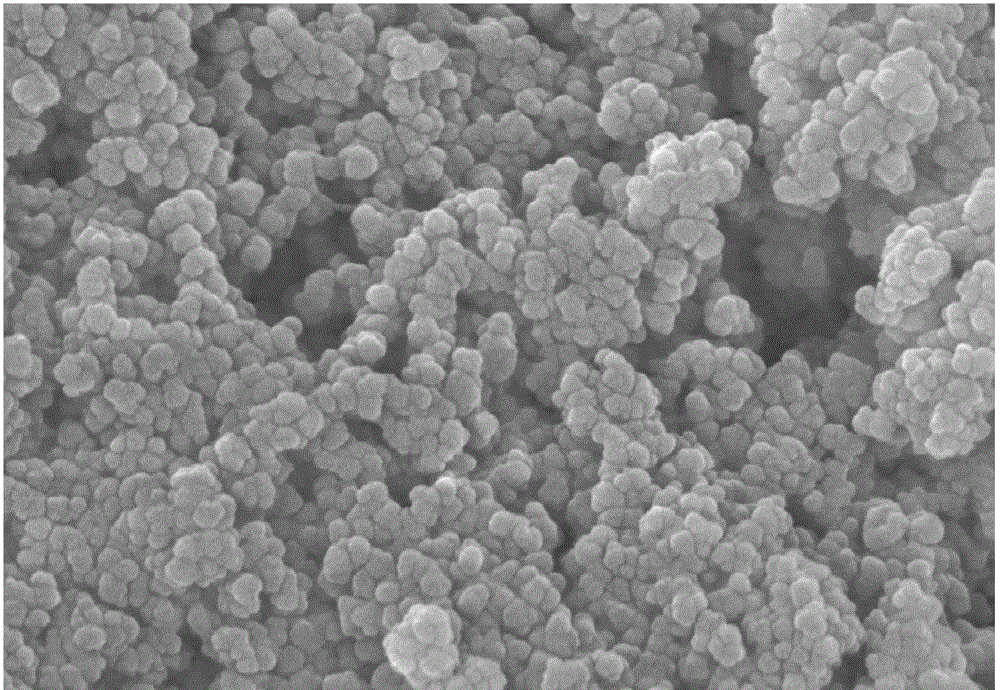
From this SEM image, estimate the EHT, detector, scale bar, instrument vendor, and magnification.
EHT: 10 kV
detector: SE(M)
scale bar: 500 nm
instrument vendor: Hitachi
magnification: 100 K X